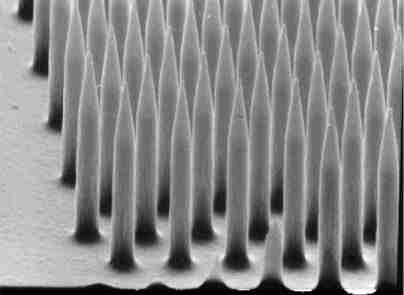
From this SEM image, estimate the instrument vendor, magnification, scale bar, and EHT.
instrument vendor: JEOL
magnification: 9 K X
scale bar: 3330 nm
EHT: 10 kV